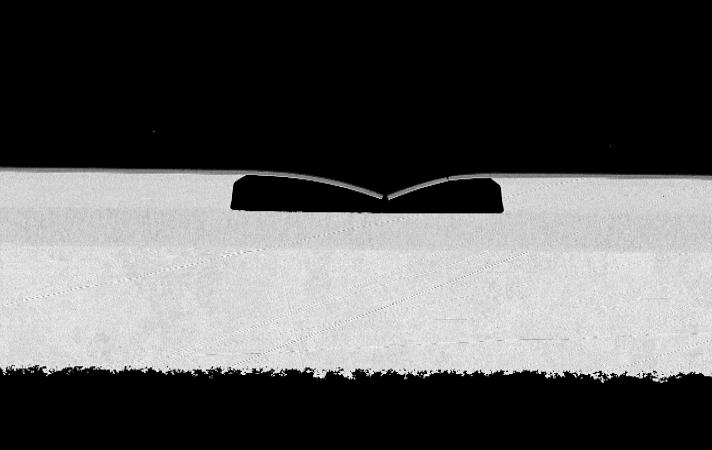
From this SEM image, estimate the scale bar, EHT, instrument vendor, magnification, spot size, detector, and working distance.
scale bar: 20000 nm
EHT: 10 kV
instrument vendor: FEI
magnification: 1 K X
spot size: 3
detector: BSE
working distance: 9.7 mm